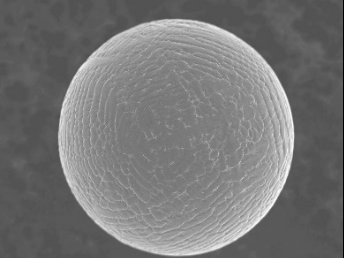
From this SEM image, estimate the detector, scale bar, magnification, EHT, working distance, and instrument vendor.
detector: SEI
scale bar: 10000 nm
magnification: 2.5 K X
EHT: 5 kV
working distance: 10 mm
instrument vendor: JEOL